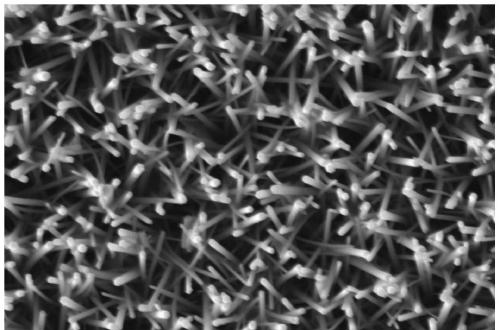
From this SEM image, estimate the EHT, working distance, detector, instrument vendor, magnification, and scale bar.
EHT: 5 kV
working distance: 11.8 mm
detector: SE2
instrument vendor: Zeiss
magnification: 50 K X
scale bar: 200 nm